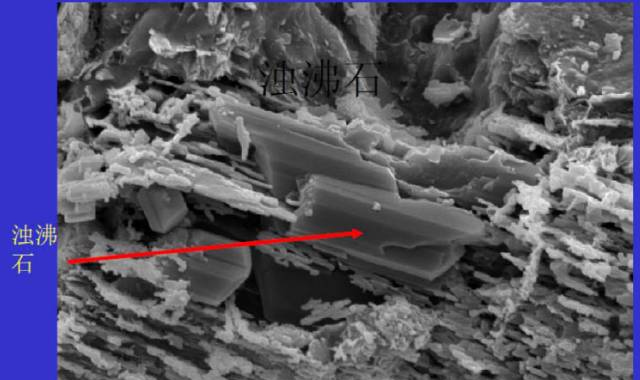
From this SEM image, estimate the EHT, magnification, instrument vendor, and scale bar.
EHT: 20 kV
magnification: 2.2 K X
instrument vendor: JEOL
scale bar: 10000 nm